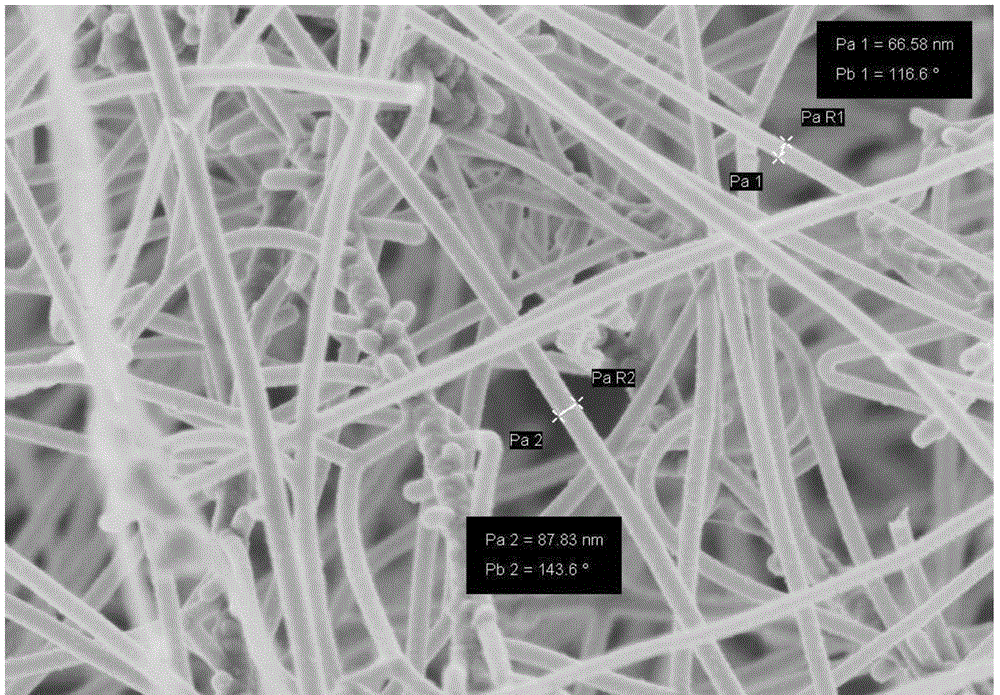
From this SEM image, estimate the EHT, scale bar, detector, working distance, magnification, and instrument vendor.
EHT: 2 kV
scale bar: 200 nm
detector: InlensDuo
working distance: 4.9 mm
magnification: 30 K X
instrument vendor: Zeiss